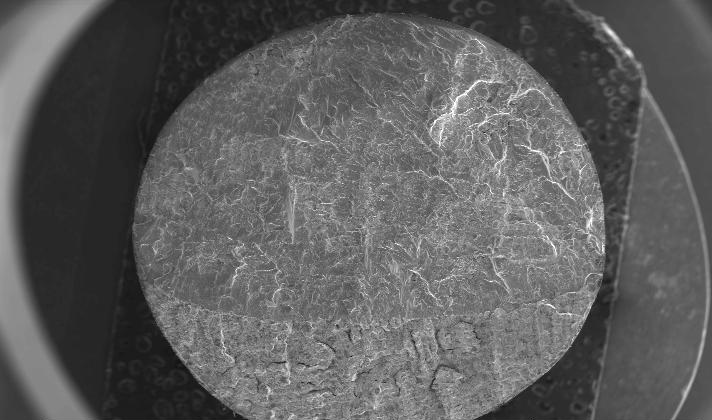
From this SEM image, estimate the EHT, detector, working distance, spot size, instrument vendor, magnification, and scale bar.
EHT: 20 kV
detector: SE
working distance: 19 mm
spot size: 5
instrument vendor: FEI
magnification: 0.01 K X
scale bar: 2000000 nm